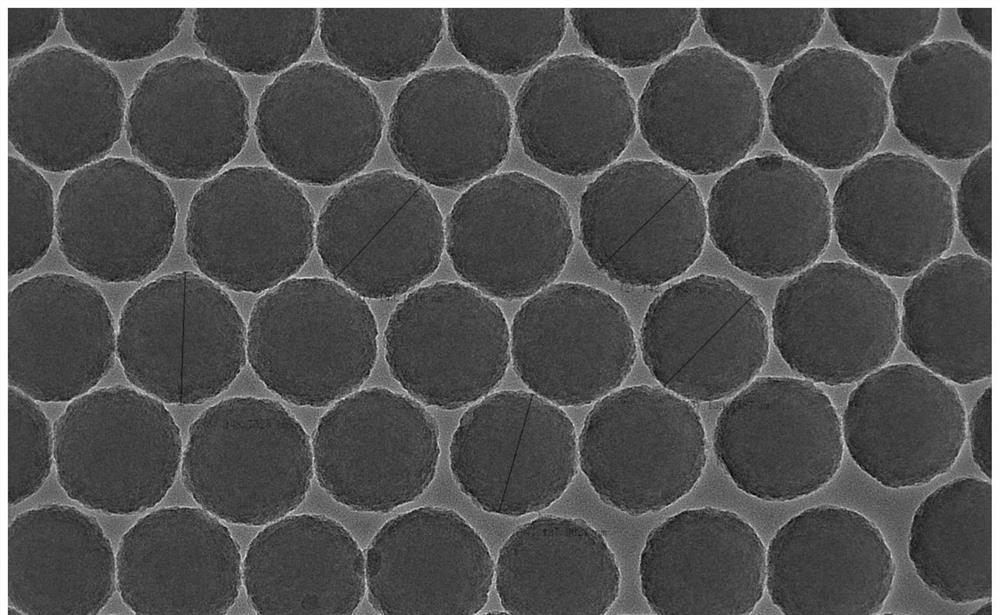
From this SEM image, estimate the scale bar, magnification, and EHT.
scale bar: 100 nm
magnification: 50 K X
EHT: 200 kV